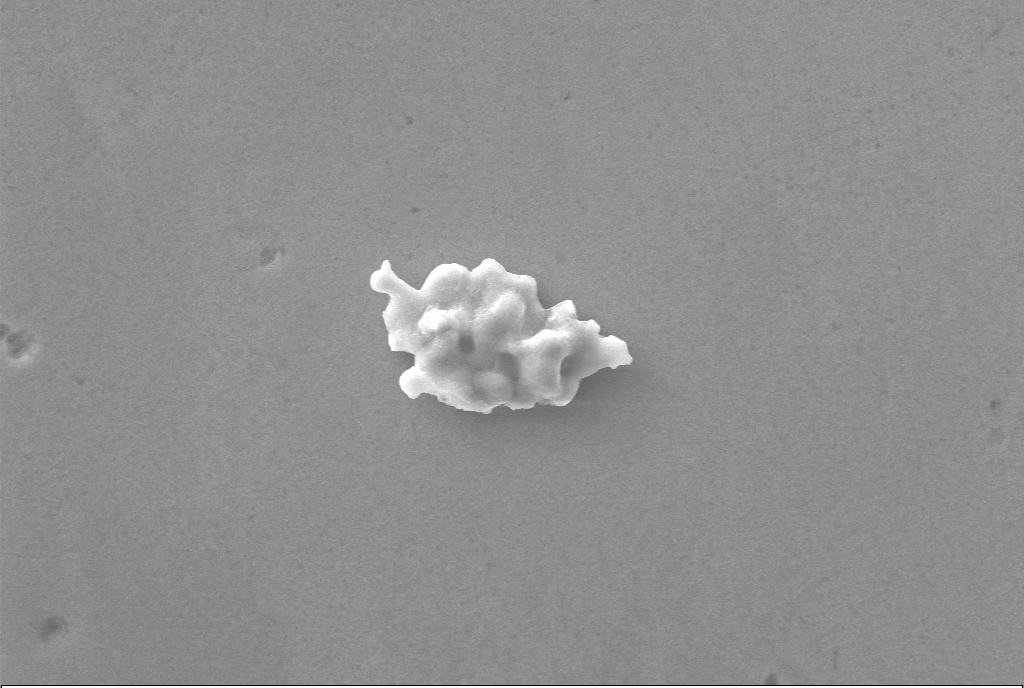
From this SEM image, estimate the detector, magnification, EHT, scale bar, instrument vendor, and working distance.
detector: SE1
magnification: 5 K X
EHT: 20 kV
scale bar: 2000 nm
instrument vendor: Zeiss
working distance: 8.5 mm